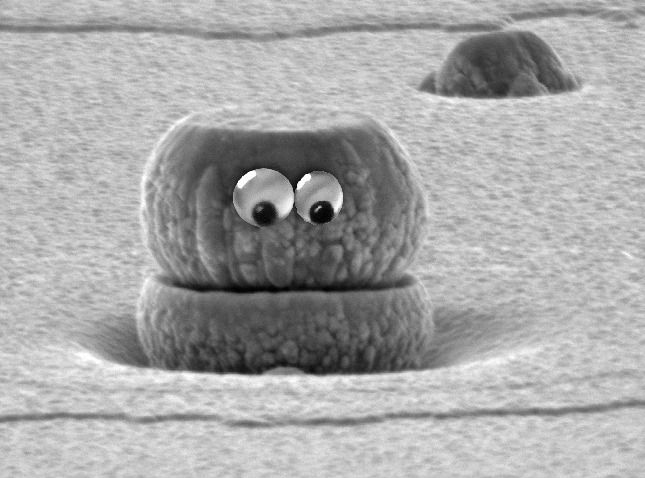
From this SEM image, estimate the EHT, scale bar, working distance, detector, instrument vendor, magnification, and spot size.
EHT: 10 kV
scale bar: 1000 nm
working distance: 17.5 mm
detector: SE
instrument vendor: FEI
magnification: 26.332 K X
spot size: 3